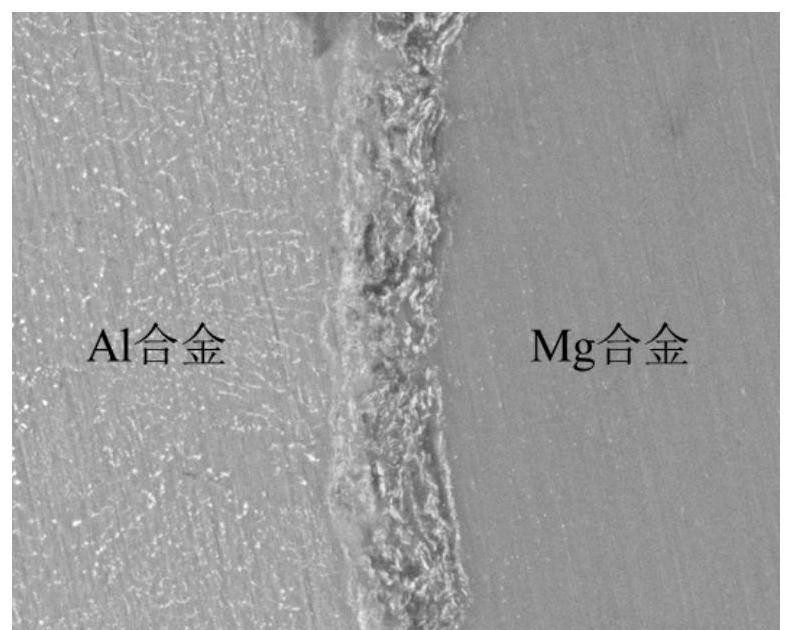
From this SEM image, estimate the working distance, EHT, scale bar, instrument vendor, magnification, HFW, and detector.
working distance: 10.6 mm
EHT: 20 kV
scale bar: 50000 nm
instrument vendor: FEI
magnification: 2 K X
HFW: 149 µm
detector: BSED